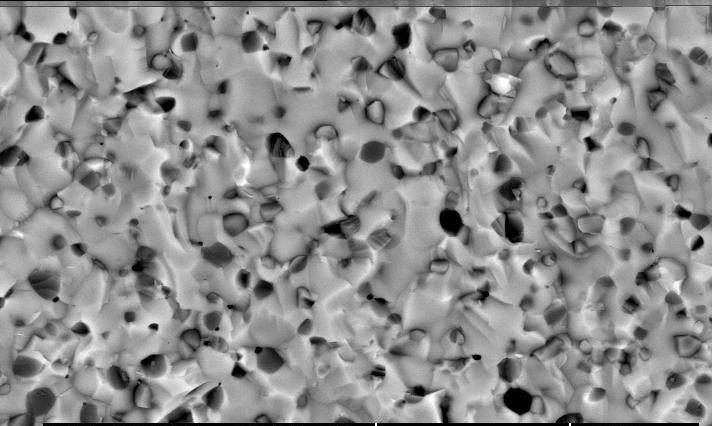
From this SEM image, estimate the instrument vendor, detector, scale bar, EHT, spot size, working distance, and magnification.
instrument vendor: FEI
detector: BSE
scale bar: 10000 nm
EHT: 20 kV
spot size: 3.6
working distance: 7.8 mm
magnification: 2.5 K X